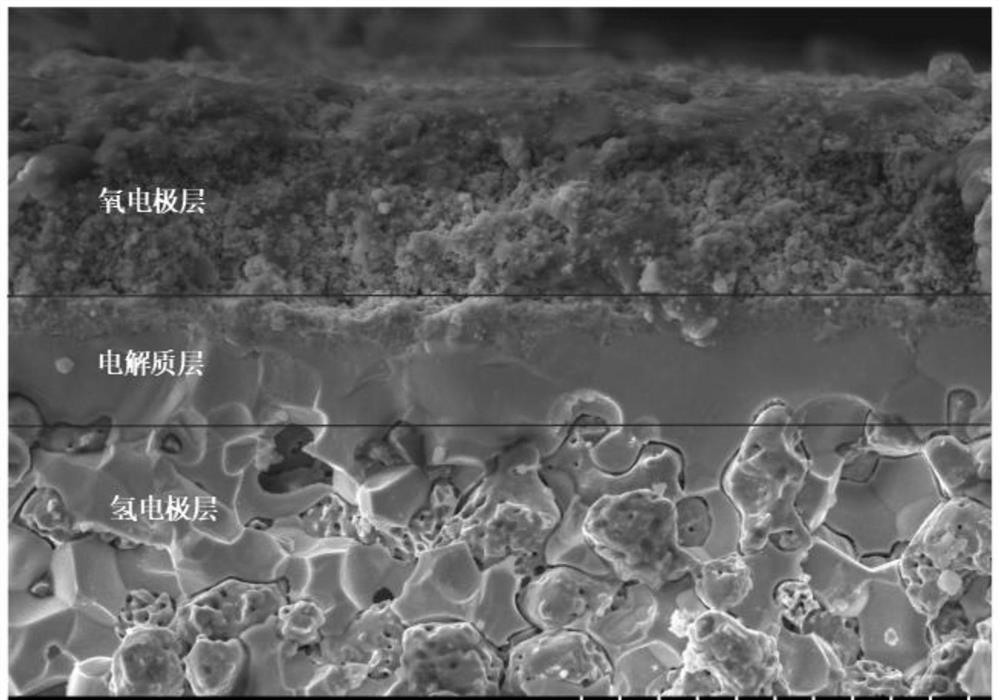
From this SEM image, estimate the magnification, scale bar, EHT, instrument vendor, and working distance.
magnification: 1 K X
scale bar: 50000 nm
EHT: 15 kV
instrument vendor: Hitachi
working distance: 9.6 mm